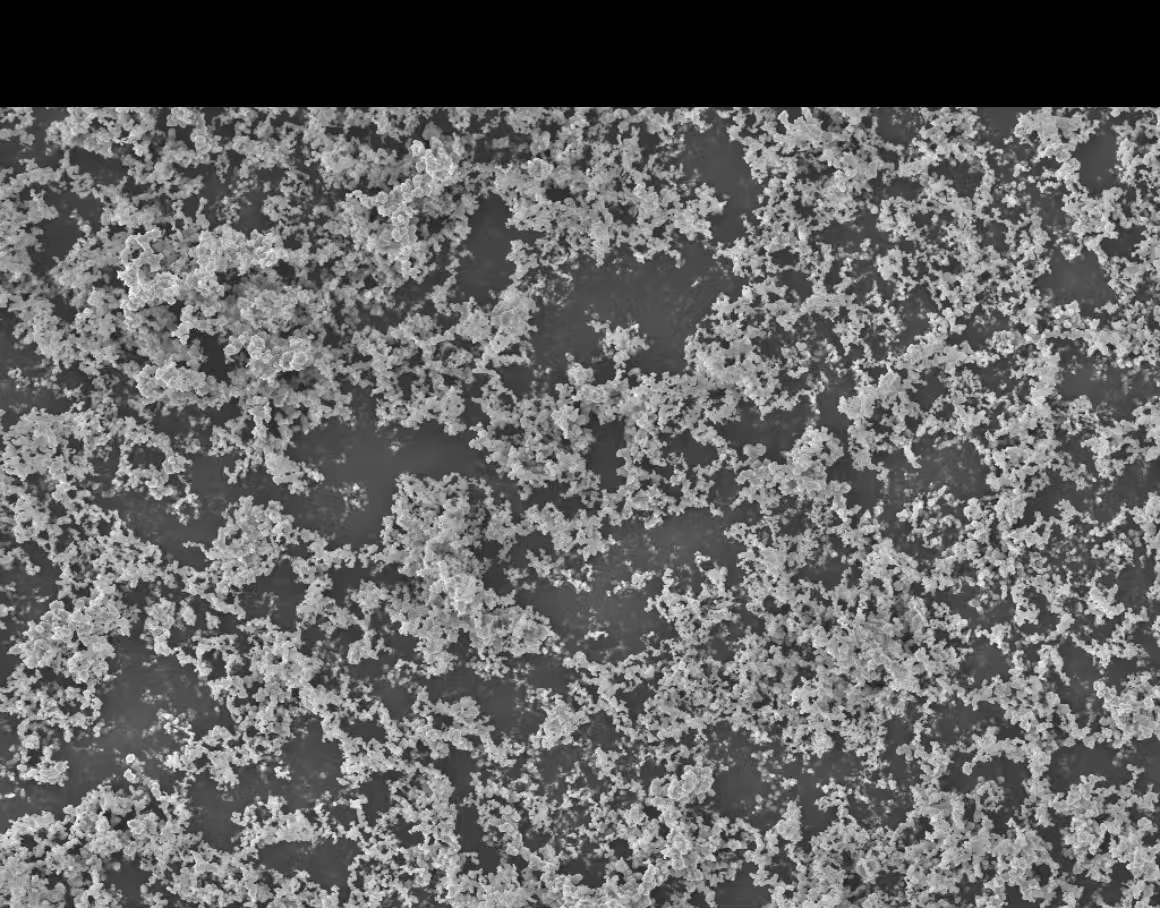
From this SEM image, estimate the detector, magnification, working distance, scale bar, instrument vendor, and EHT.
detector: InLens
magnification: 2 K X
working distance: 4.1 mm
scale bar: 4000 nm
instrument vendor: Zeiss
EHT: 5 kV